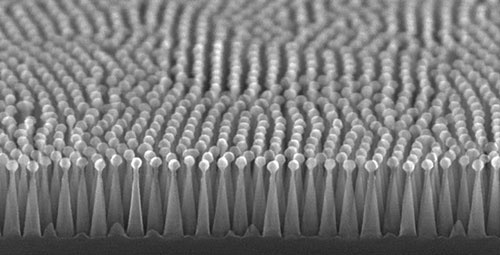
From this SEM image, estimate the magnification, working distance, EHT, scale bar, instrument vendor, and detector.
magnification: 100 K X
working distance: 5.7 mm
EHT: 20 kV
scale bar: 500 nm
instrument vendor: Hitachi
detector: SE(U,LA0)